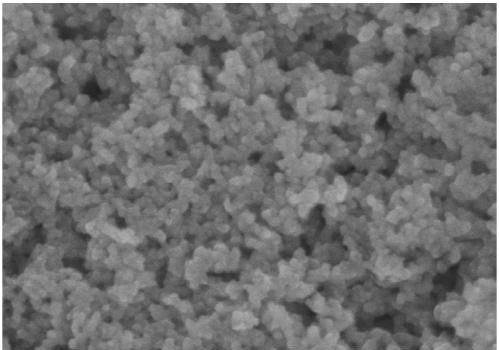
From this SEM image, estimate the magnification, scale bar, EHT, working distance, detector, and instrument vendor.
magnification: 100 K X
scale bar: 500 nm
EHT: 5 kV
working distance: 8 mm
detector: SE(U)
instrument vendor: Hitachi